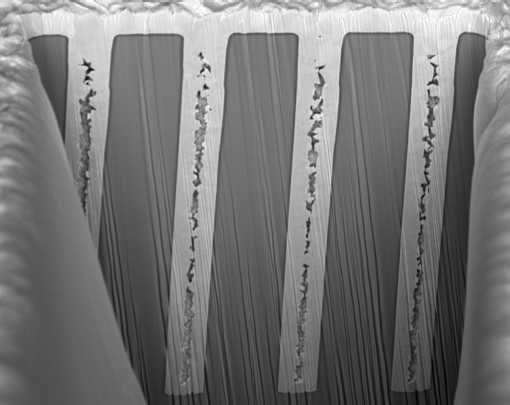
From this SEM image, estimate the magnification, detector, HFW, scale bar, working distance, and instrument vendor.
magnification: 1.75 K X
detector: TLD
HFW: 85.3 µm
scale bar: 20000 nm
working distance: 4 mm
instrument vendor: FEI